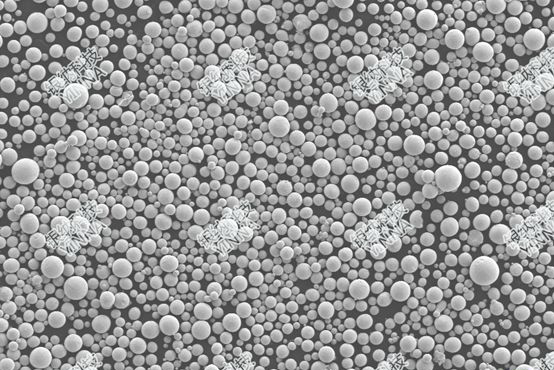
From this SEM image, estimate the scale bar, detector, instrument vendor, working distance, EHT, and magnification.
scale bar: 20000 nm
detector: SE2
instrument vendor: Zeiss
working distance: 7.6 mm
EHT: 5 kV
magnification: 0.2 K X